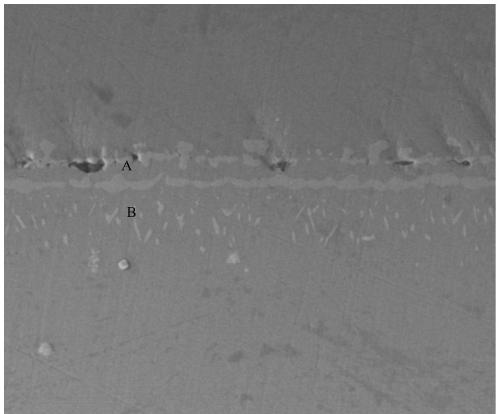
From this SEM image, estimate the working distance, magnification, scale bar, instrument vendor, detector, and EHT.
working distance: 11.9 mm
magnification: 5 K X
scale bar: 30000 nm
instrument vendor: FEI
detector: ETD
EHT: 10 kV